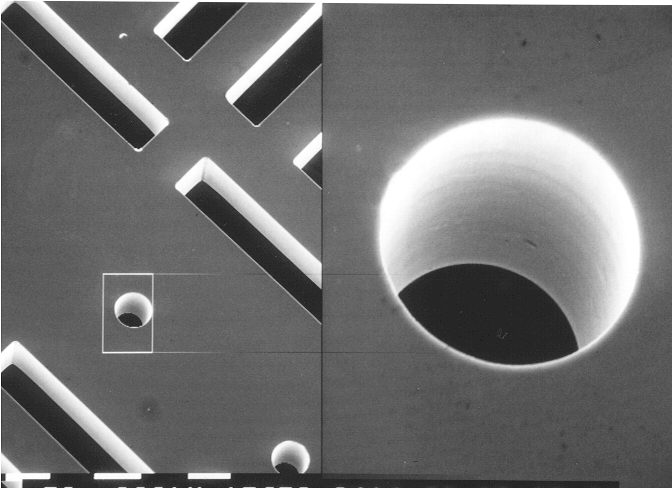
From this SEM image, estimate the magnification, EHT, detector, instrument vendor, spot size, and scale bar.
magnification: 0.156 K X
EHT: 29.9 kV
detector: SE/SE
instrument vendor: FEI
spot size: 7.4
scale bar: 50000 nm